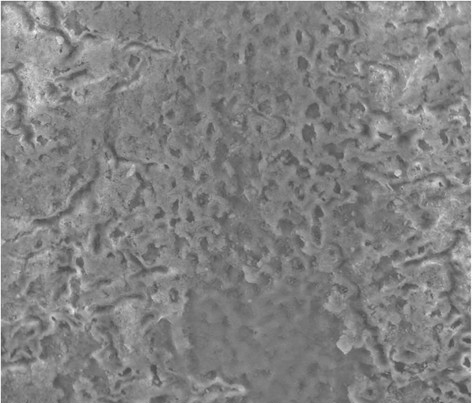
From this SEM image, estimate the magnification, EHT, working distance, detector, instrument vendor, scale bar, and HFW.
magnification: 2 K X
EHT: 20 kV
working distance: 10 mm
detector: LFD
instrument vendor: FEI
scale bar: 50000 nm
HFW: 149 µm